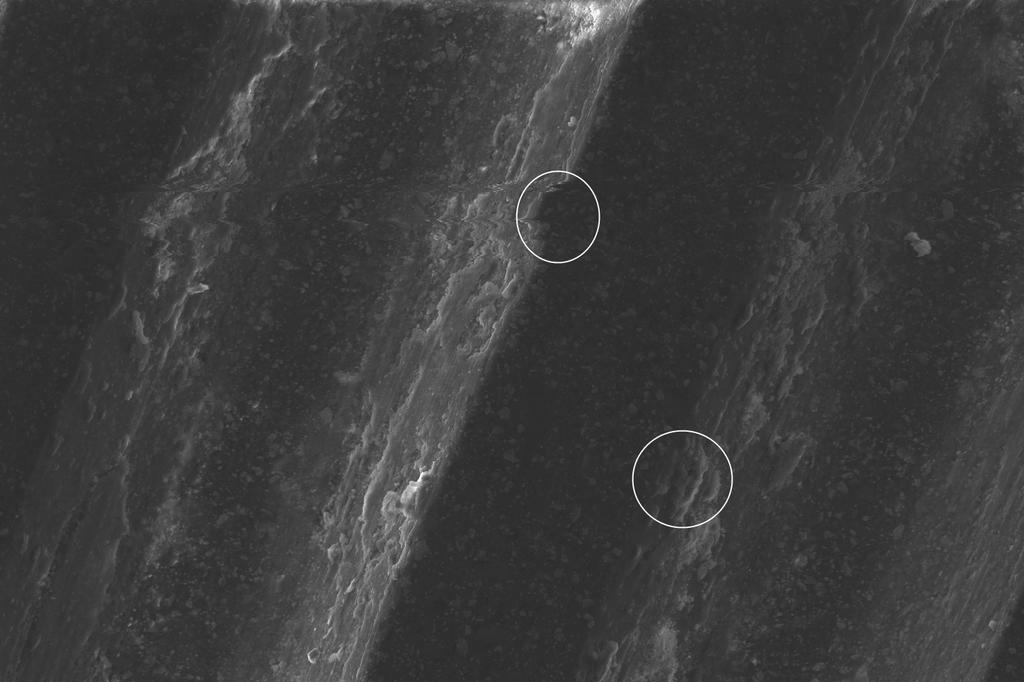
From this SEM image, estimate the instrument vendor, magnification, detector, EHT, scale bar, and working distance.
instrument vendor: FEI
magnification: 0.8 K X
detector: ETD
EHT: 20 kV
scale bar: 100000 nm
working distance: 13.6 mm